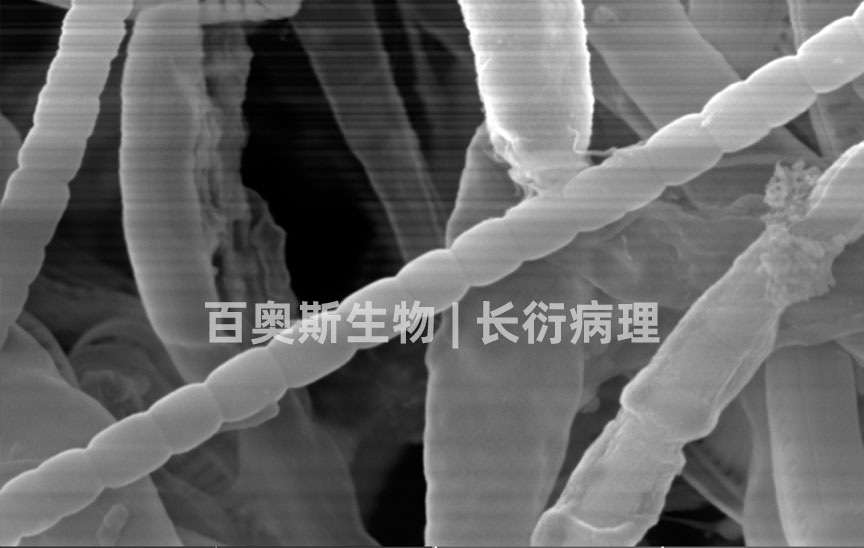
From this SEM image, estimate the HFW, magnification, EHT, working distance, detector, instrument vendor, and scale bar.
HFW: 19.1 µm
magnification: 10 K X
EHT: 20 kV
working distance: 10.15 mm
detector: SE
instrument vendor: Tescan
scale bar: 5000 nm